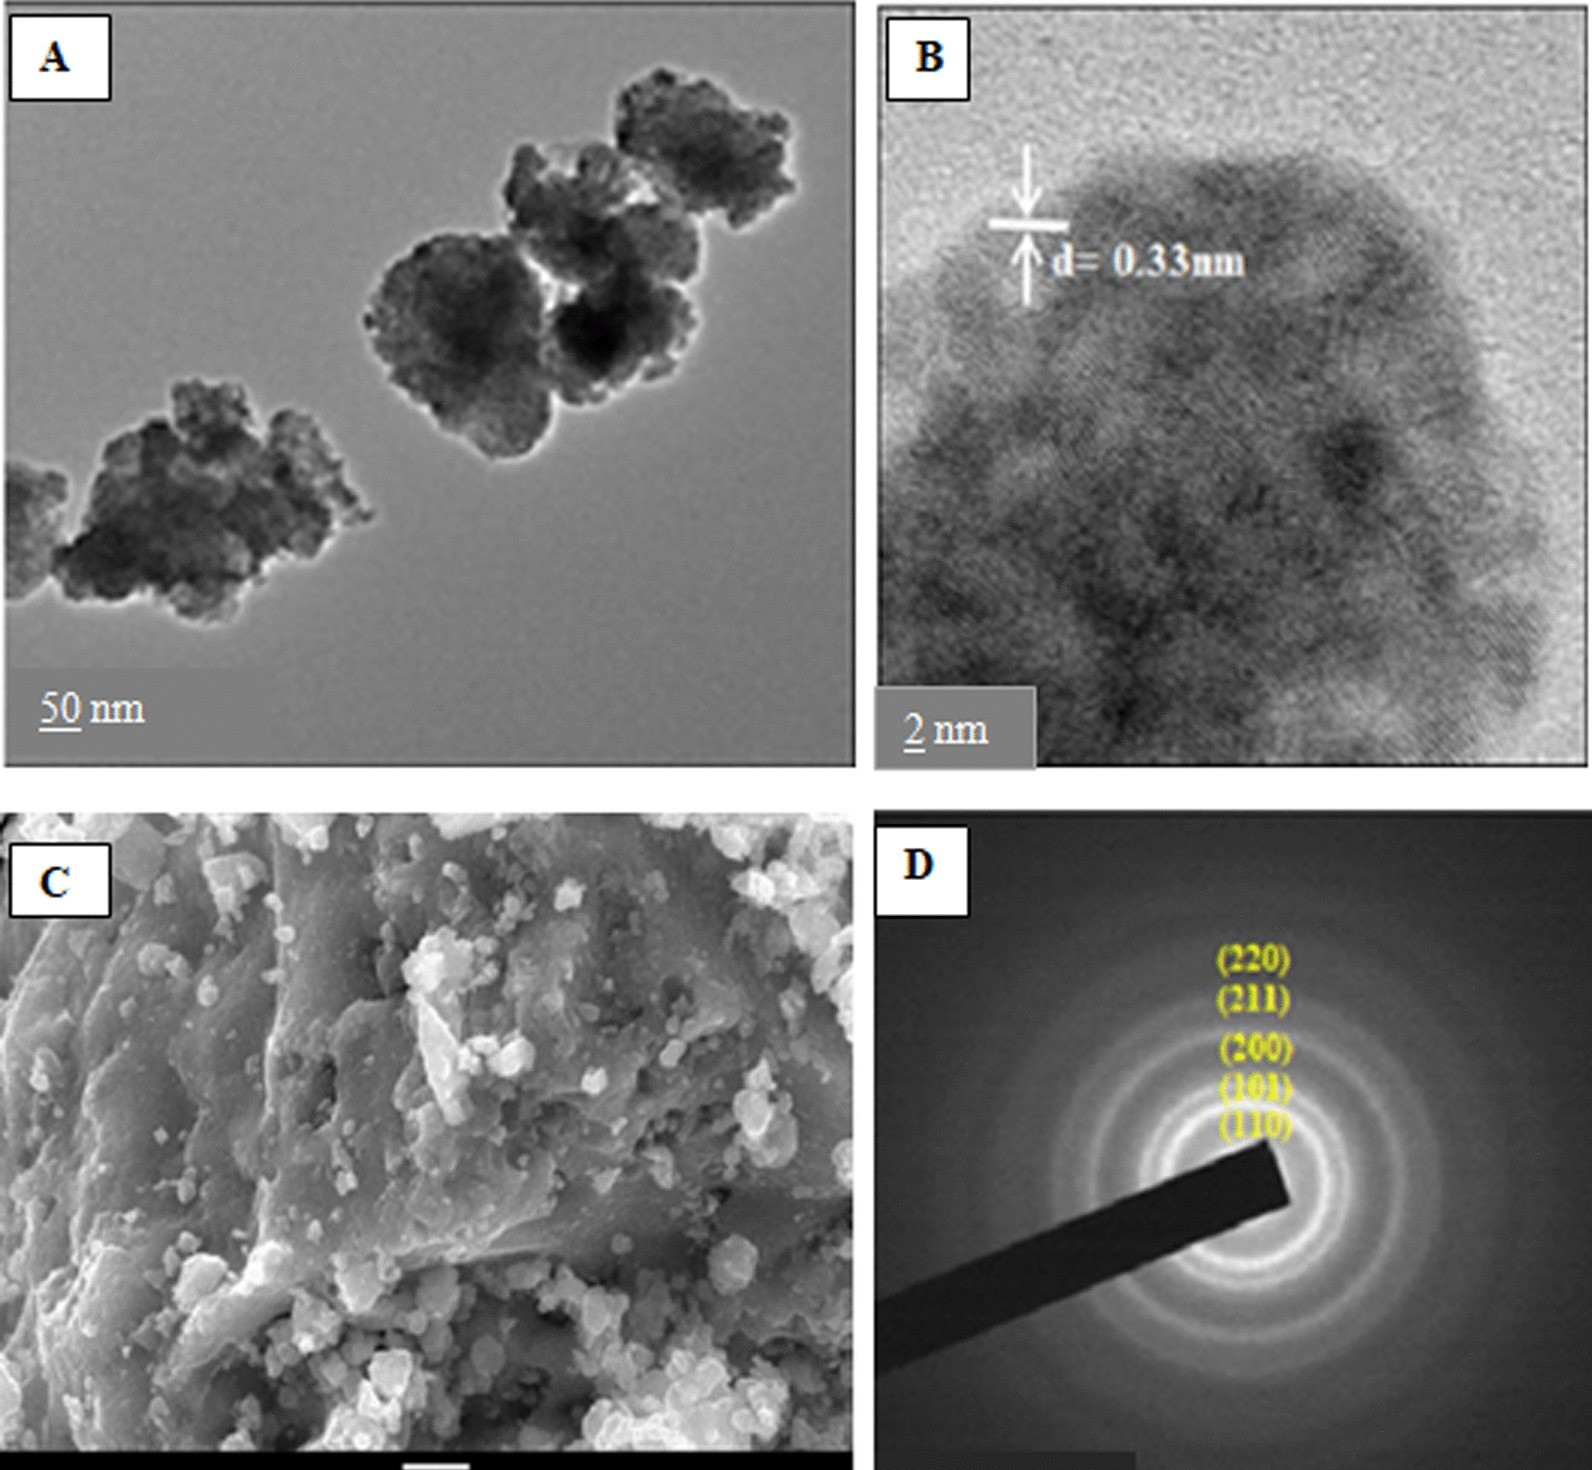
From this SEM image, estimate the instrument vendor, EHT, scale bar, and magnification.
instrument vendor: JEOL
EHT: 20 kV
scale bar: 1000 nm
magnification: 10 K X